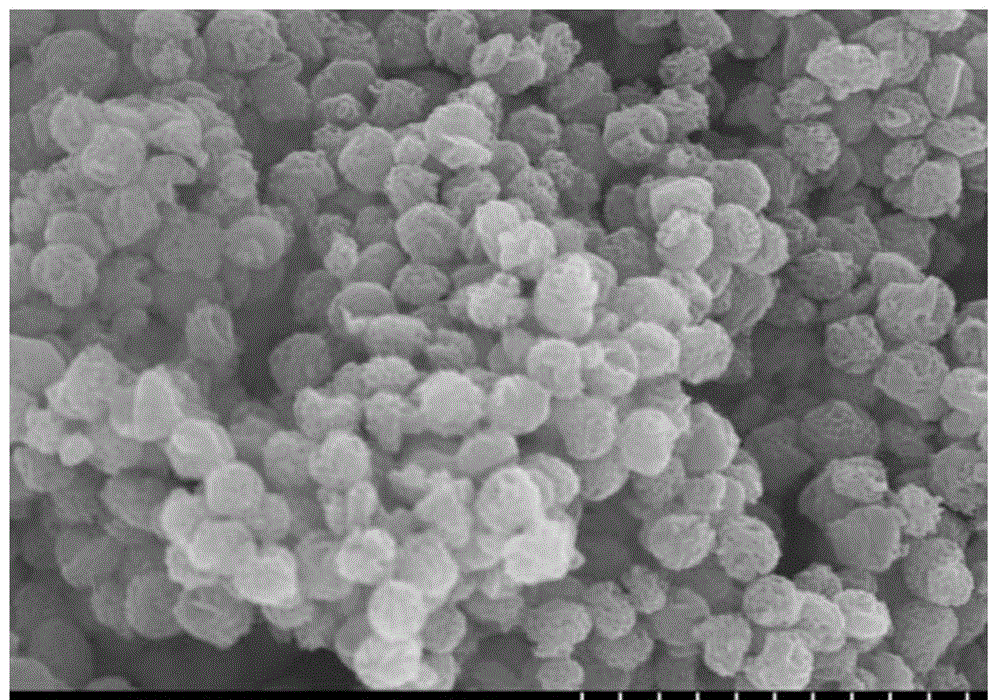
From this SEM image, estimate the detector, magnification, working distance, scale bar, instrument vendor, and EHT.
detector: SE(M)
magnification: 25 K X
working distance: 8.5 mm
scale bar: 2000 nm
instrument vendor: Hitachi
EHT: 10 kV